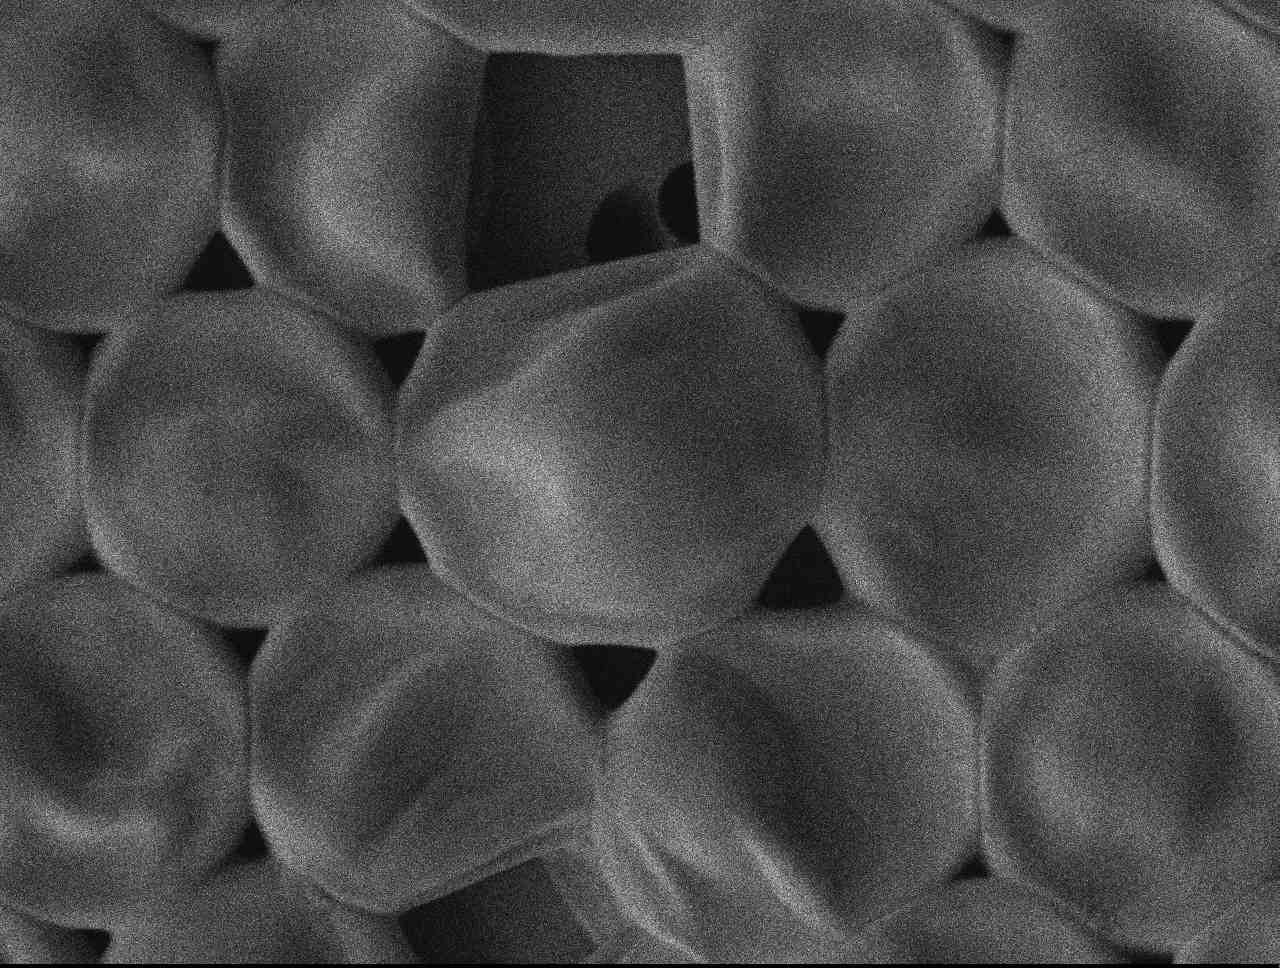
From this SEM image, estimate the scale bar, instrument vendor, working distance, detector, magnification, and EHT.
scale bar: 1000 nm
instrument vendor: JEOL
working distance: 8 mm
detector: LEI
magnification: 7.5 K X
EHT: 1.5 kV